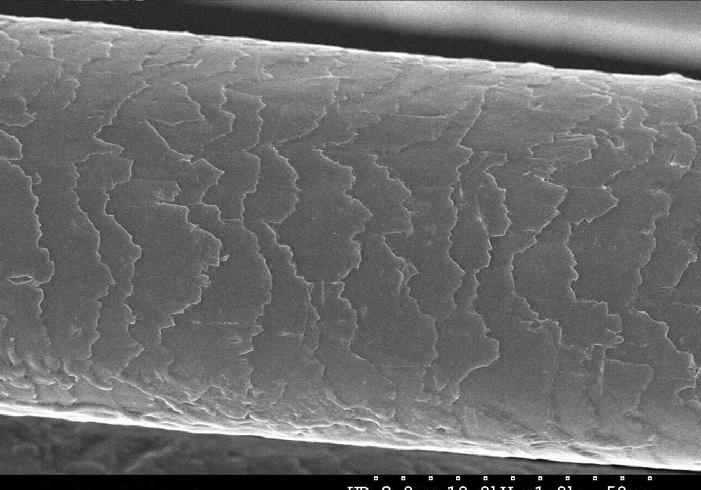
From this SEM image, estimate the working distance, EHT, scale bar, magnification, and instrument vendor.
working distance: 8 mm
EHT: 10 kV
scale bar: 50000 nm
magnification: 1 K X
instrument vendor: Hitachi